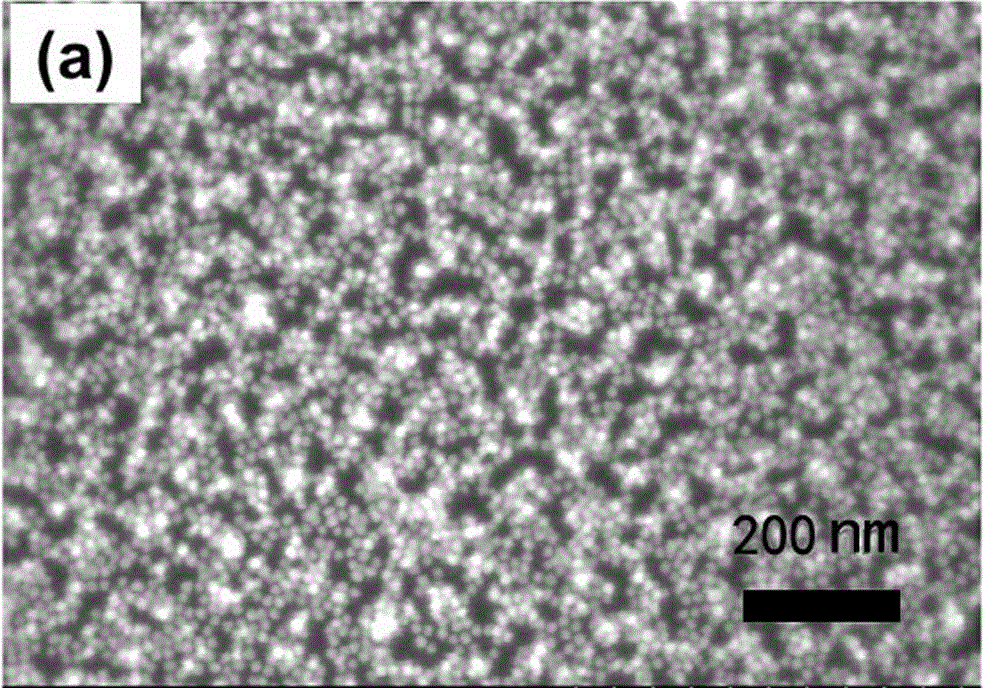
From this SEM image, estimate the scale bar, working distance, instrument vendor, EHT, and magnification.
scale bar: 500 nm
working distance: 7.8 mm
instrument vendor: Hitachi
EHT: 5 kV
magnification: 100 K X